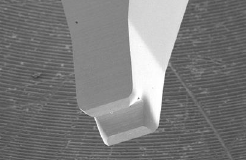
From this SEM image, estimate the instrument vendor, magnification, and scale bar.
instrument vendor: Hitachi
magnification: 0.05 K X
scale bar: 500000 nm